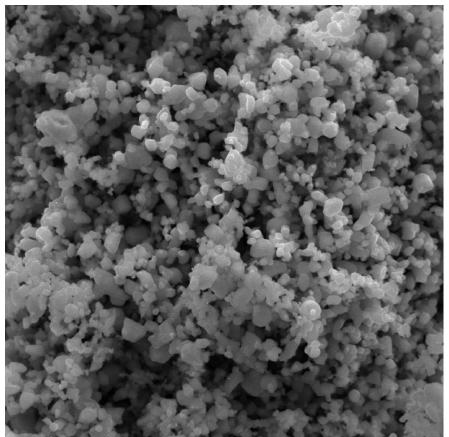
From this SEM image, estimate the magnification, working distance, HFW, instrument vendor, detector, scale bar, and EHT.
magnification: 10 K X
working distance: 9.85 mm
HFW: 20.8 µm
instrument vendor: Tescan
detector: SE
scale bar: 5000 nm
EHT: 20 kV